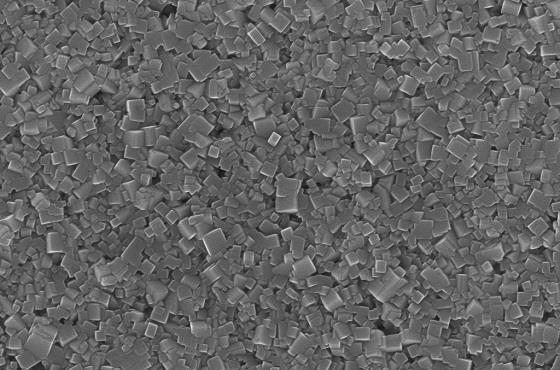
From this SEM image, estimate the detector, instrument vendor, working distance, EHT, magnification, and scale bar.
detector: InLens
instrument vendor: Zeiss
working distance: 8.7 mm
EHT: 15 kV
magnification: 20 K X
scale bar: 200 nm